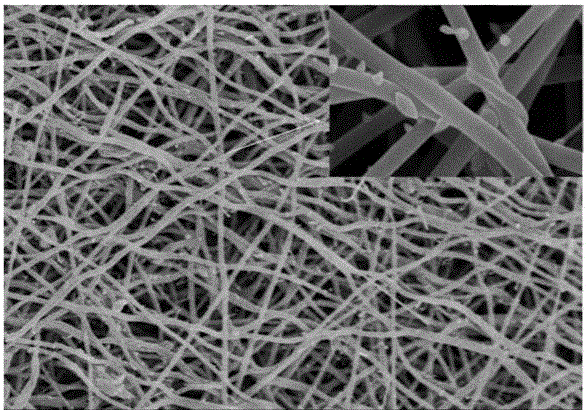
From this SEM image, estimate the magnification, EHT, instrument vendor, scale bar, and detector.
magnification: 10 K X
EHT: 5 kV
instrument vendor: Hitachi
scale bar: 5000 nm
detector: SE(M)